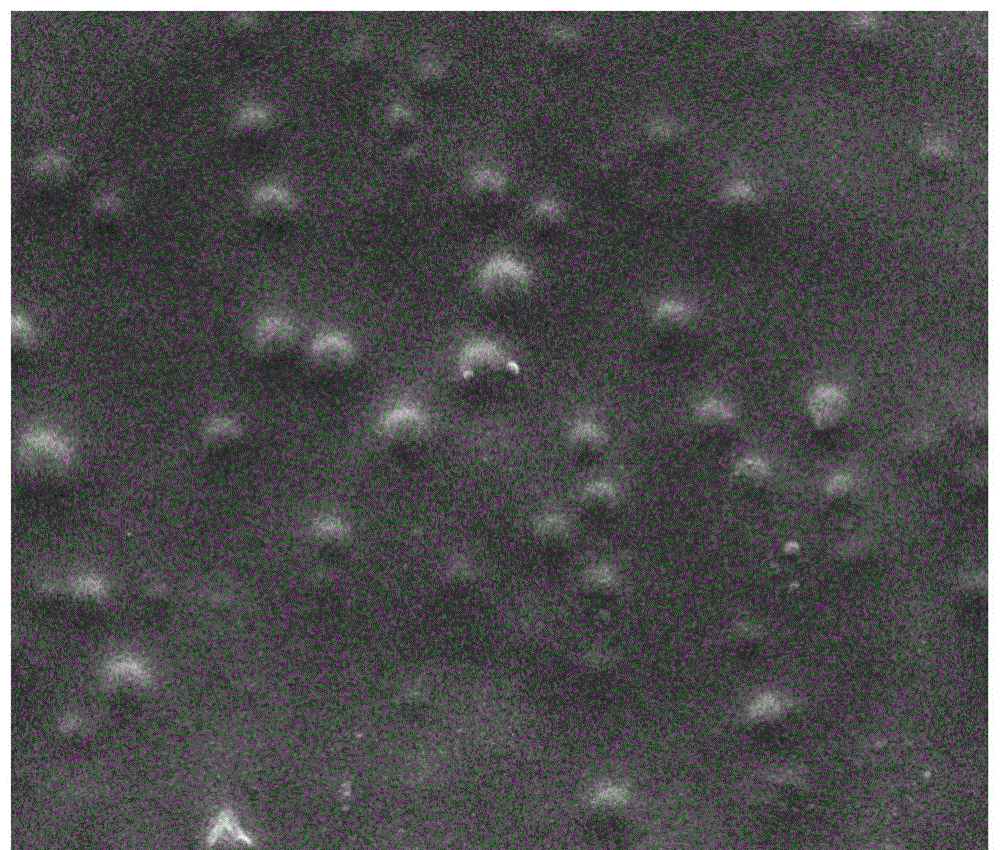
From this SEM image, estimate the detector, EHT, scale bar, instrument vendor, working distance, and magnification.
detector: ETD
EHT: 20 kV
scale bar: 5000 nm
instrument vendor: FEI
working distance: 10.2 mm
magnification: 20 K X